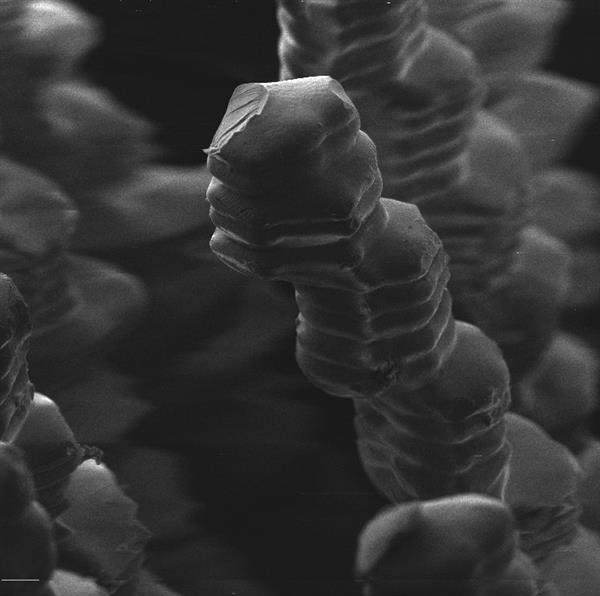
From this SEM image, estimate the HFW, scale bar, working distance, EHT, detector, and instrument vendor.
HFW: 319 µm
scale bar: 50000 nm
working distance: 5.66 mm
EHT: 20 kV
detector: SE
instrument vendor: Tescan